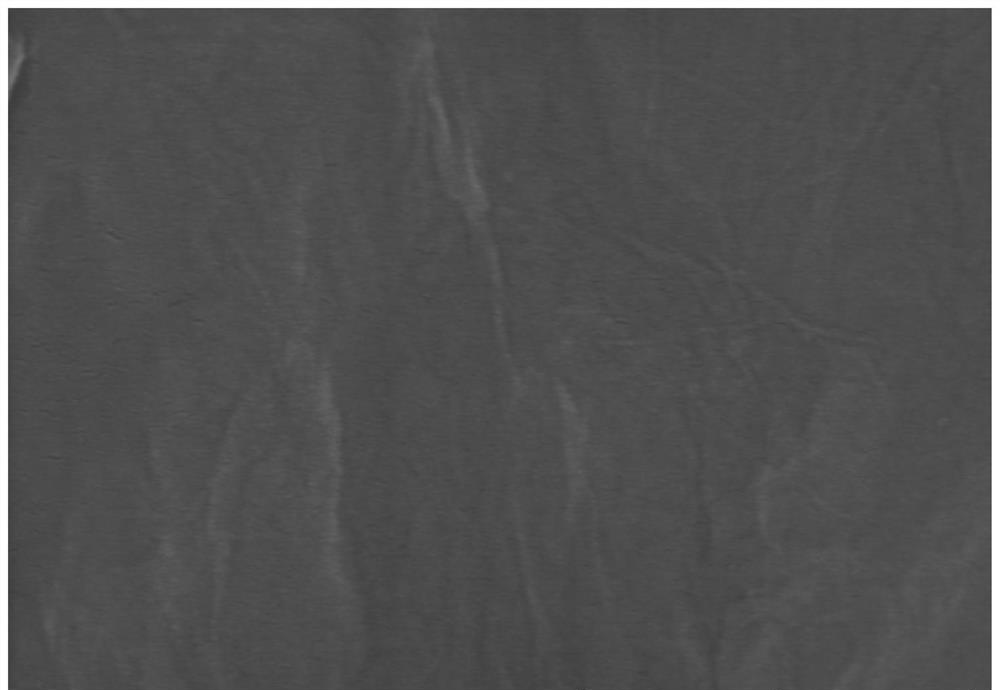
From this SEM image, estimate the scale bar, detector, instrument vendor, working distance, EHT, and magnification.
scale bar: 500 nm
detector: SE(UL)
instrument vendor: Hitachi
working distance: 9 mm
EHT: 15 kV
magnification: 100 K X